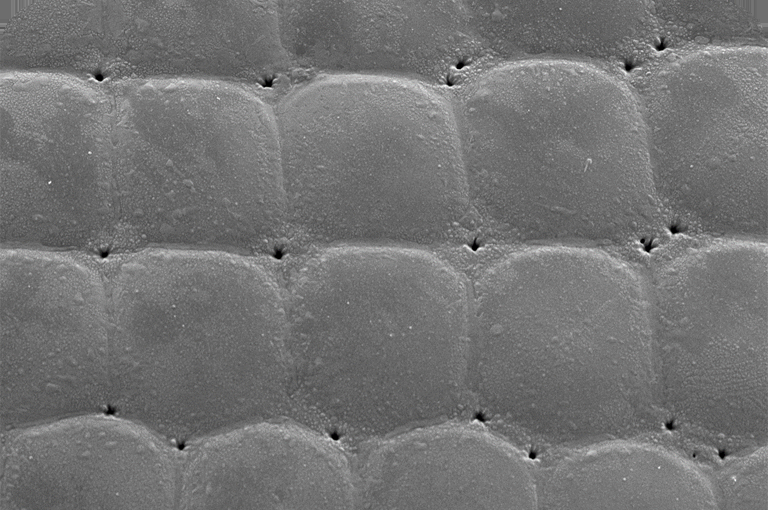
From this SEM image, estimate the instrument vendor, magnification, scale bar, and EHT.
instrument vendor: JEOL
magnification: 1 K X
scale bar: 10000 nm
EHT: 10 kV